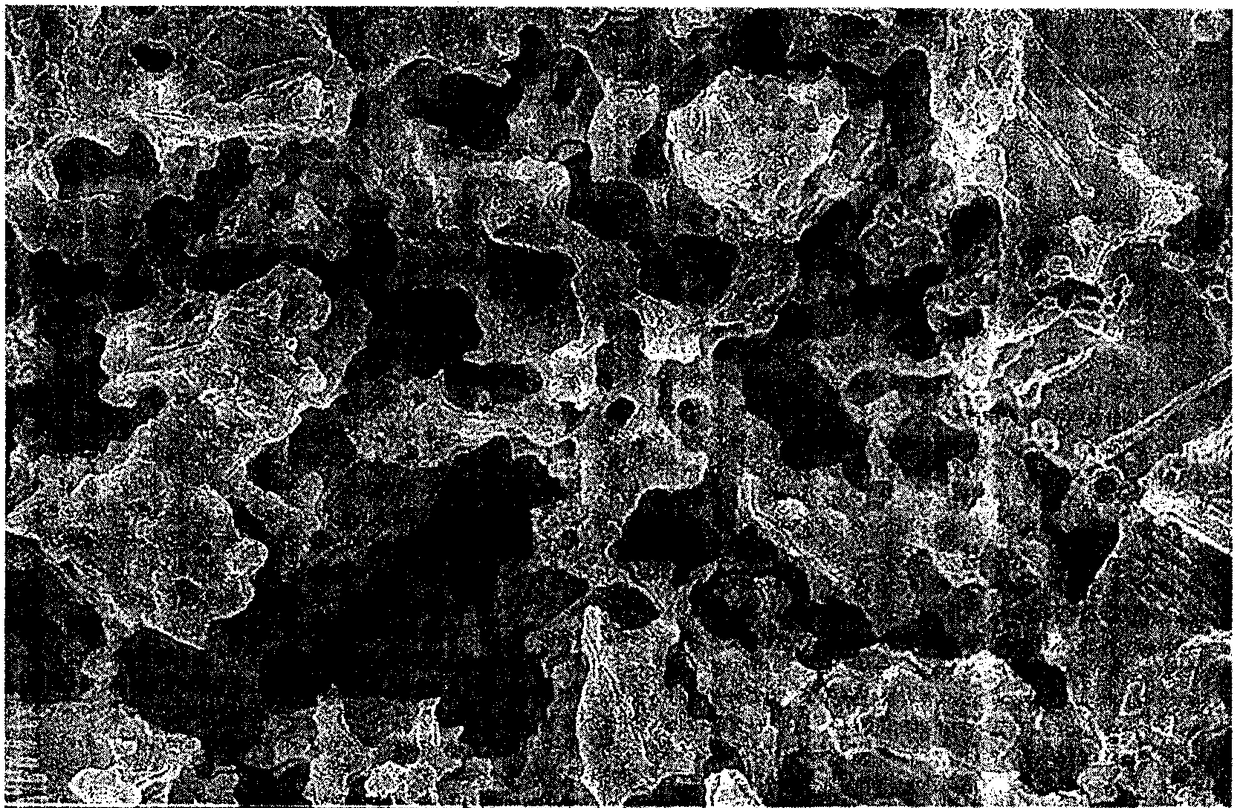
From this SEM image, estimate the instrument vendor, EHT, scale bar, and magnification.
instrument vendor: Hitachi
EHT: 15 kV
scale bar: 20000 nm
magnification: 2 K X